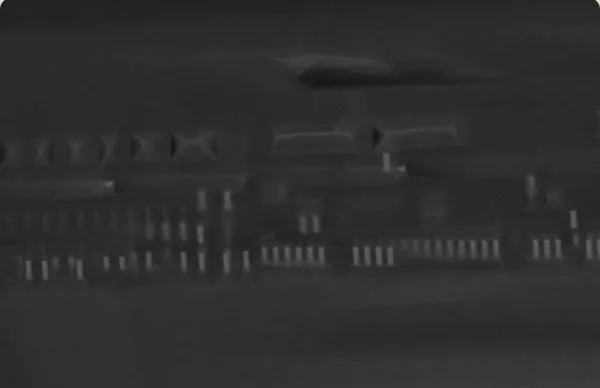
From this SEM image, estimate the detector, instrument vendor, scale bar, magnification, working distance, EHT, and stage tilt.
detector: InLens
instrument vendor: Zeiss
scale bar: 2000 nm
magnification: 11.47 K X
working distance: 4 mm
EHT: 15 kV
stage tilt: -15°